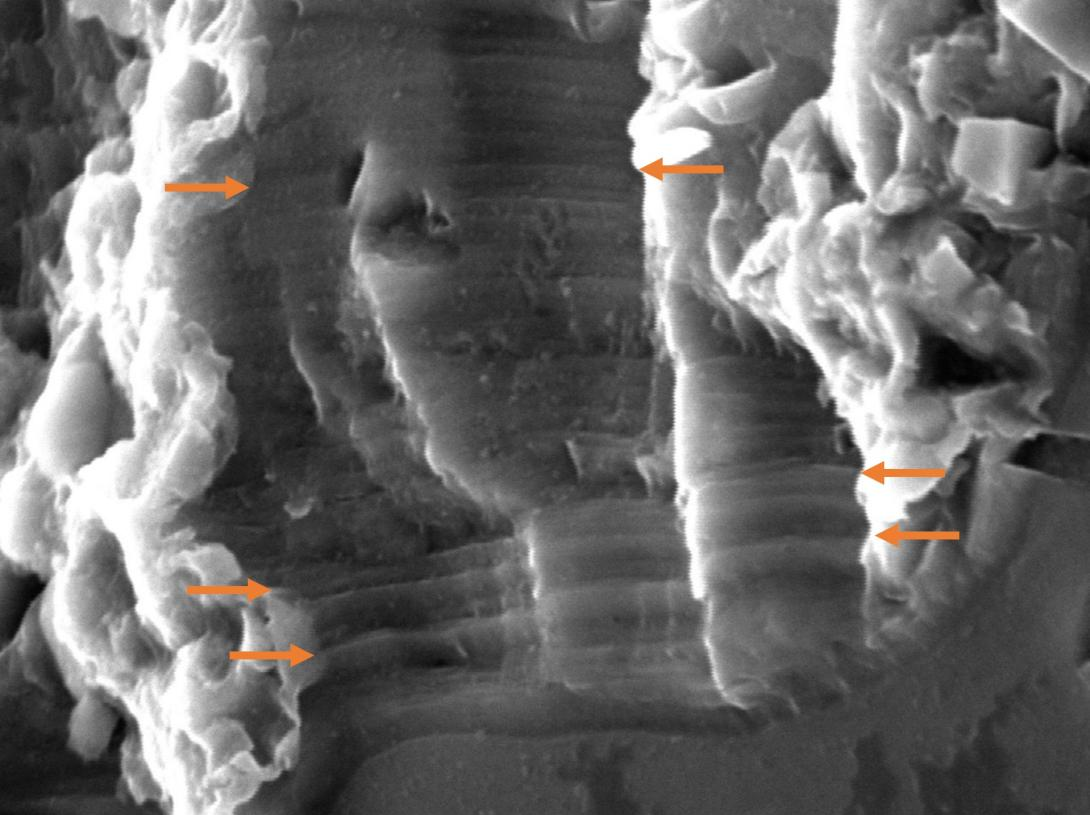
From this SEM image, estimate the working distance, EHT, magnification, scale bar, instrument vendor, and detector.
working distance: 18.1 mm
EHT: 20 kV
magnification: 4.5 K X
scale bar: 5000 nm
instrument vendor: JEOL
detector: SED